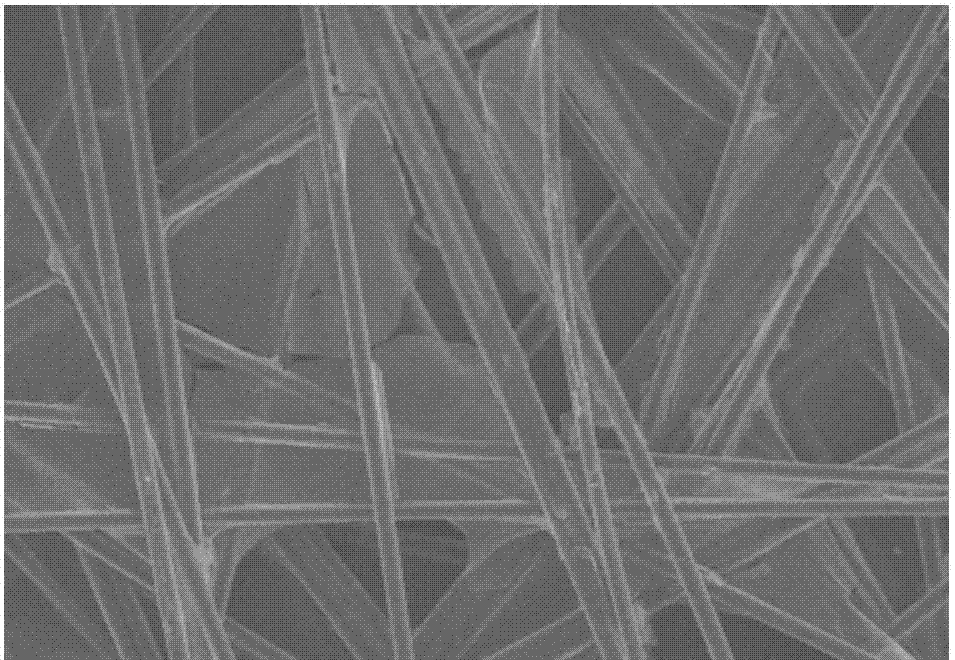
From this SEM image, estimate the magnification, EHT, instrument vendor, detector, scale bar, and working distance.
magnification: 0.35 K X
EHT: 15 kV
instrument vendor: Hitachi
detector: SE(UL)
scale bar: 100000 nm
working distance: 10.8 mm